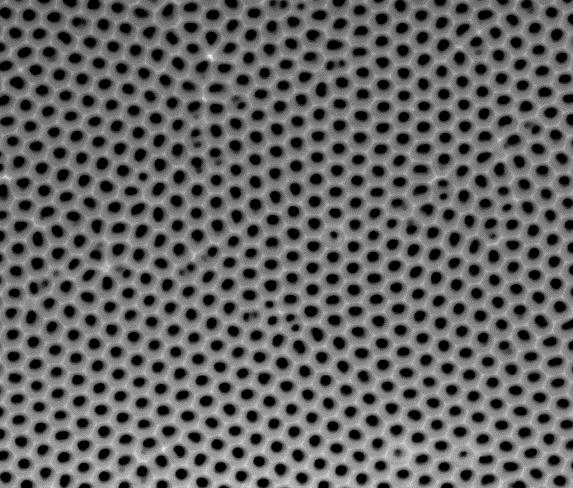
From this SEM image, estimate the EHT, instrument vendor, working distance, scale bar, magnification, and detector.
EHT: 15 kV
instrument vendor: FEI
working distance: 9.5 mm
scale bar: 1000 nm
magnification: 100 K X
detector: ETD